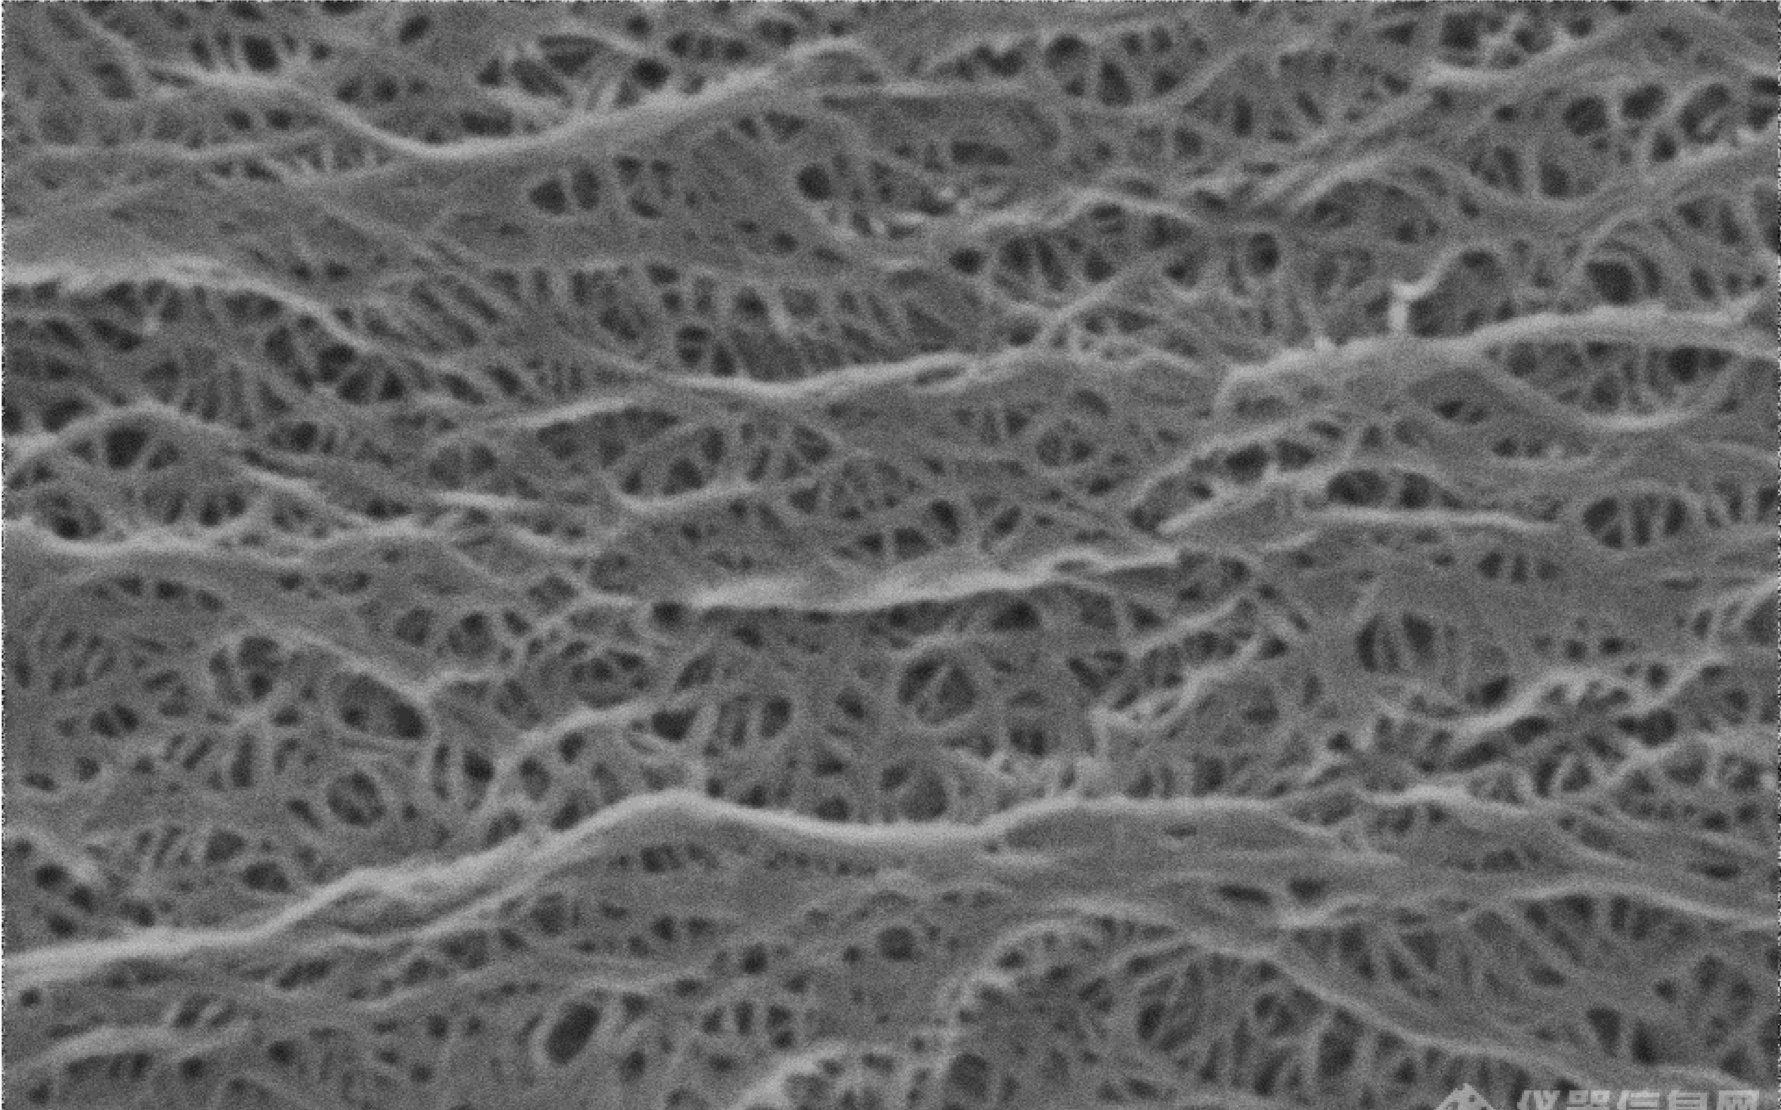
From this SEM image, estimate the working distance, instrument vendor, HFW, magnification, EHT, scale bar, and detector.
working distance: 6.902 mm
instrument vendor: Thermo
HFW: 5.19 µm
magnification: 100 K X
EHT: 10 kV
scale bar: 2000 nm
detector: Mix 34%